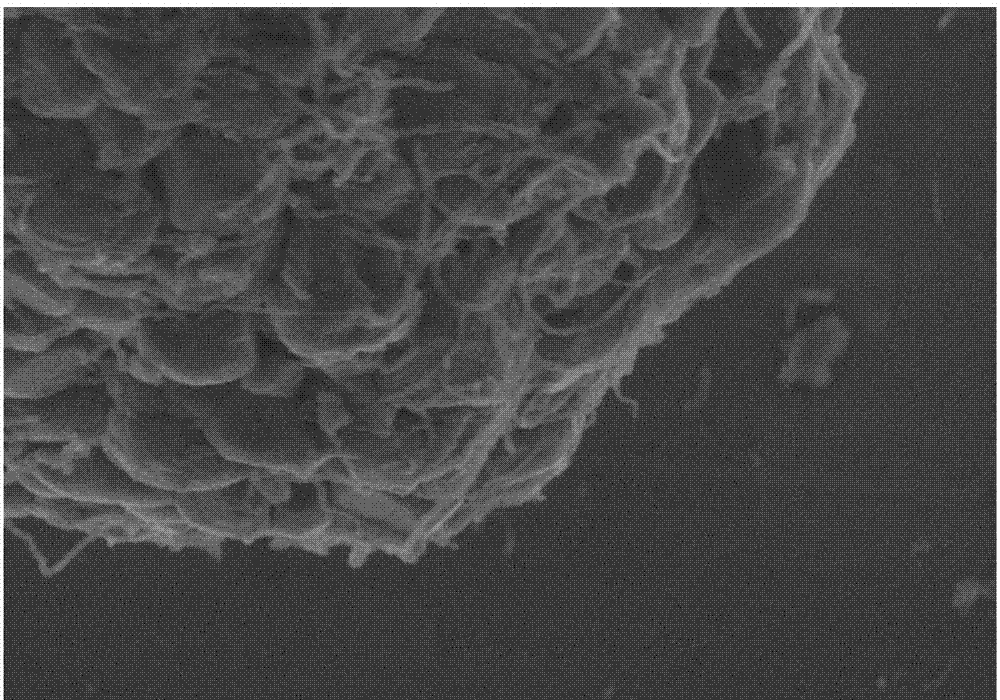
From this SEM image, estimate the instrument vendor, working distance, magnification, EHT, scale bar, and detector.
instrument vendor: Hitachi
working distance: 5.5 mm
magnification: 30 K X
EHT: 3 kV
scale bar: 1000 nm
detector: SE(UL)